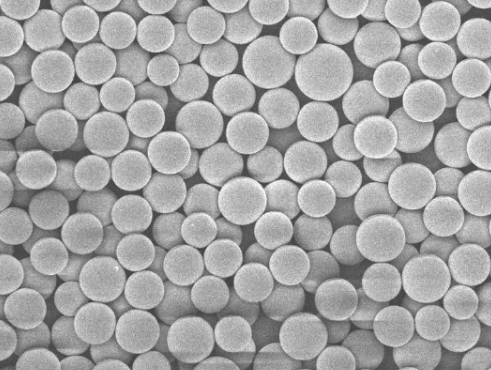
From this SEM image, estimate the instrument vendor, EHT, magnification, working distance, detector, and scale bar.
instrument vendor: JEOL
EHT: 5 kV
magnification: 2 K X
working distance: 8.3 mm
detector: SEI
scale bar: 10000 nm